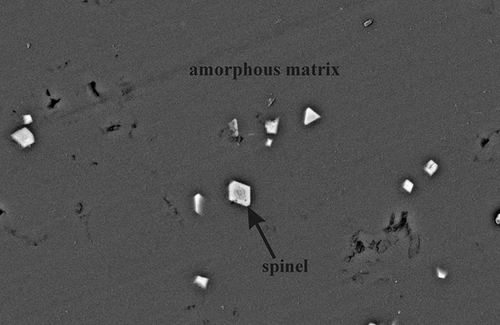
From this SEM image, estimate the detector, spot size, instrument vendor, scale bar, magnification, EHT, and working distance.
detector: BSE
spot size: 3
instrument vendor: FEI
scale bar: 10000 nm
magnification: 5 K X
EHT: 15 kV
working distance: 6.8 mm